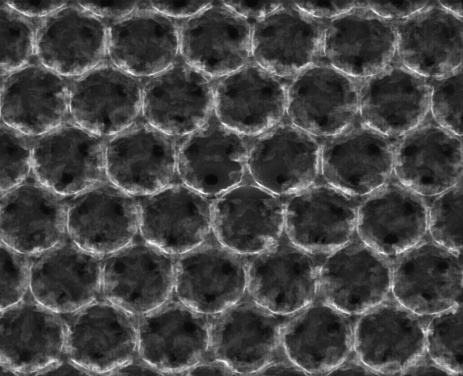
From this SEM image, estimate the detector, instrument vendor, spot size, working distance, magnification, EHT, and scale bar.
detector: SE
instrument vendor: FEI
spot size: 3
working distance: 10 mm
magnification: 100 K X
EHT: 20 kV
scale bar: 1000 nm